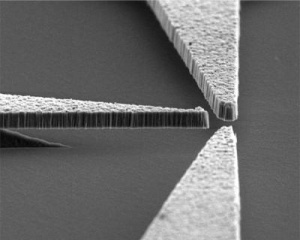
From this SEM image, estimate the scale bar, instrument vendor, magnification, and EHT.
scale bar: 7500 nm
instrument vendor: Hitachi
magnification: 4 K X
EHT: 5 kV